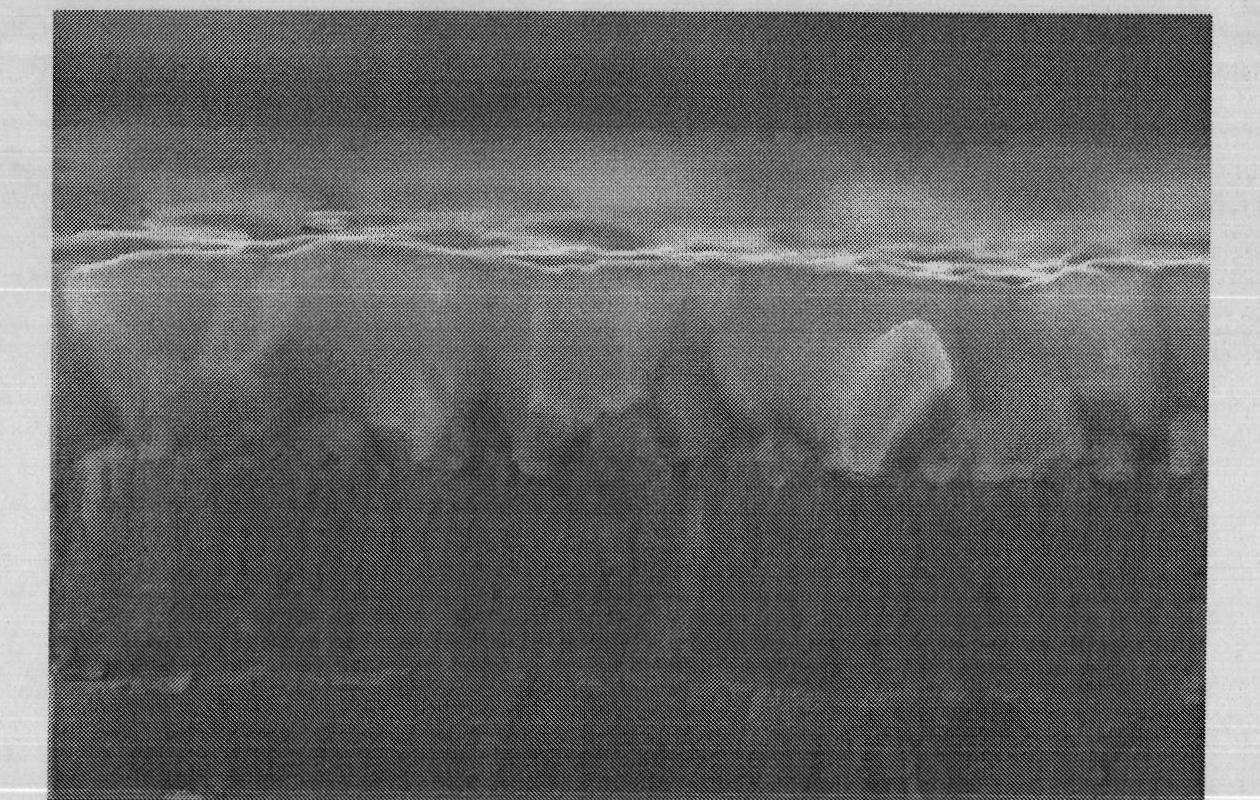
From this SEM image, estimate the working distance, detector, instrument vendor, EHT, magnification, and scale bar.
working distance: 8.6 mm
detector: SE(M)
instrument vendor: Hitachi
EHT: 15 kV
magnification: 50 K X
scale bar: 1000 nm